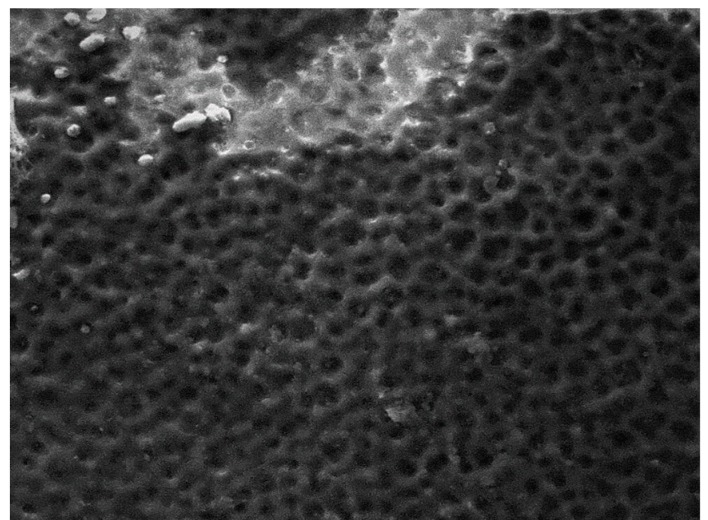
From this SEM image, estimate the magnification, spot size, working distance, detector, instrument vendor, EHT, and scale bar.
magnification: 2 K X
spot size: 5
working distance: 21.9 mm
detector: ETD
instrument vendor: FEI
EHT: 20 kV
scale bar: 50000 nm